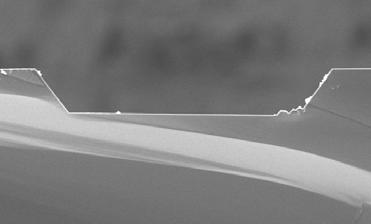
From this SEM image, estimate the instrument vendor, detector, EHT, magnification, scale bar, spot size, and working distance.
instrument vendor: FEI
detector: SE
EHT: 15 kV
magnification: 0.25 K X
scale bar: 100000 nm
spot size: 3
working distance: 10.1 mm